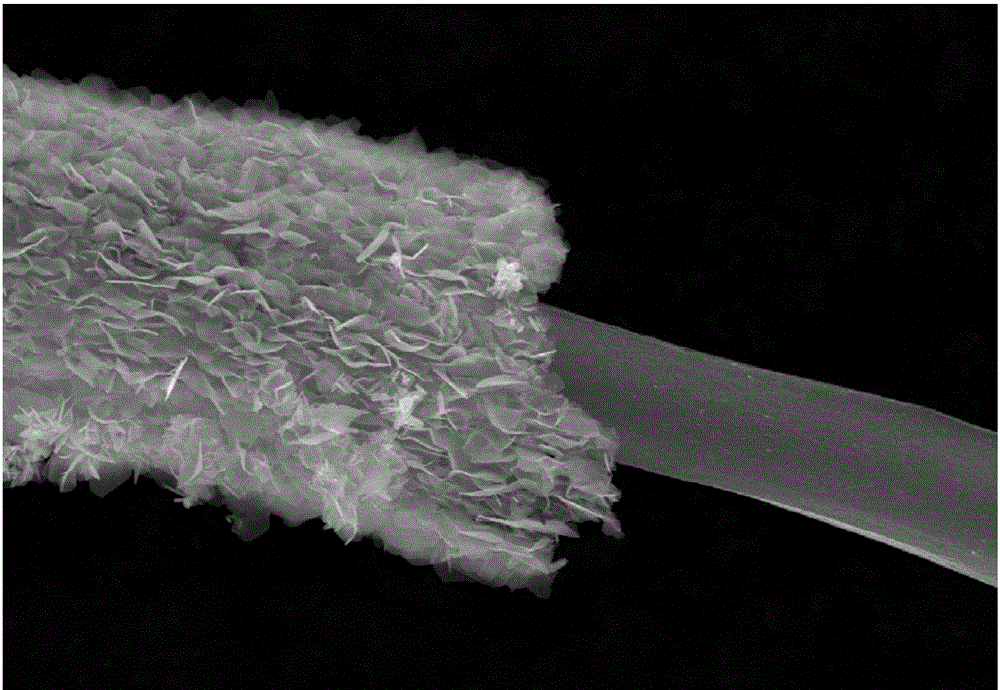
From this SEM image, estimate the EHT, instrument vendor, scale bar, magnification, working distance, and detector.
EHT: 15 kV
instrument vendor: Hitachi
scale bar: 10000 nm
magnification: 5 K X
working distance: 15.1 mm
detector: SE(M)